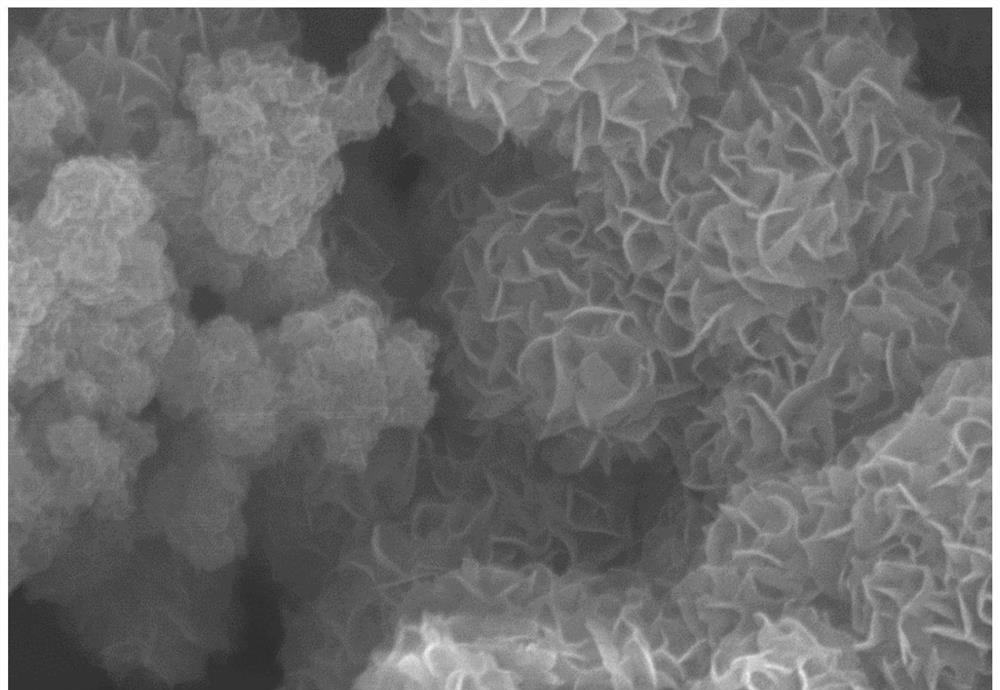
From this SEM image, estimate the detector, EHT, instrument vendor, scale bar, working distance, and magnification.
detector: SE(U)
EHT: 10 kV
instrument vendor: Hitachi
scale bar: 1000 nm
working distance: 10.6 mm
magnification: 50 K X